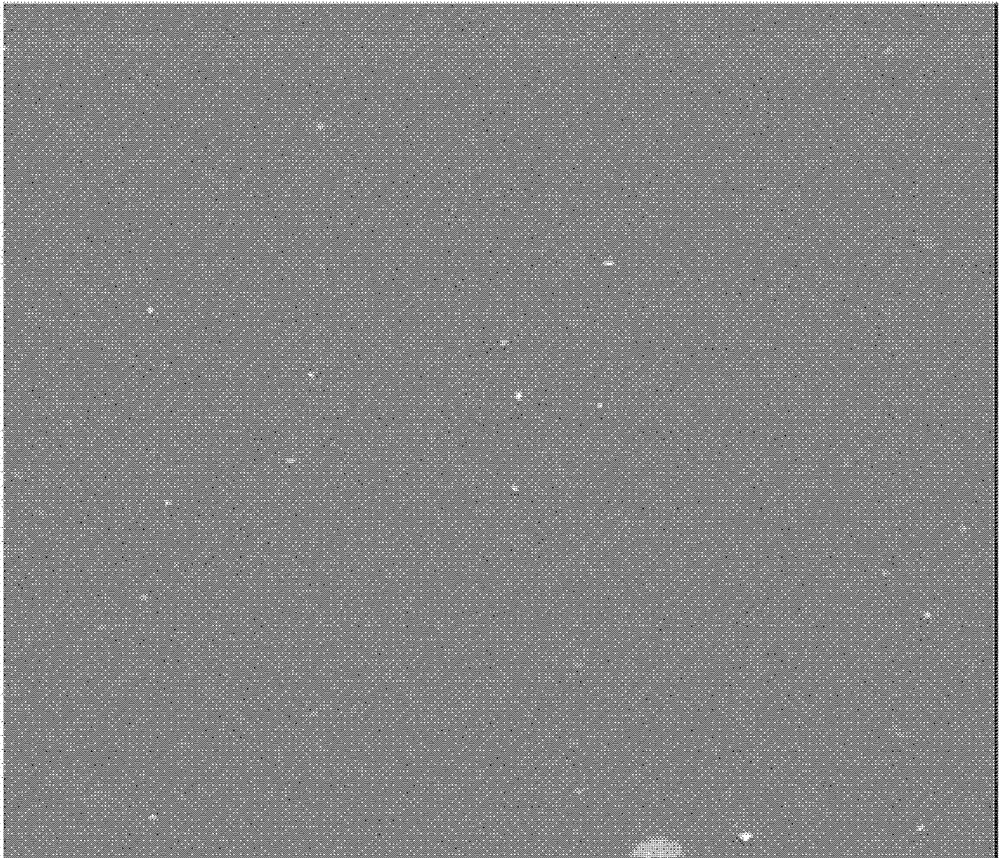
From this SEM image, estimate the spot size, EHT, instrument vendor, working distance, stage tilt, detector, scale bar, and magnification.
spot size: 4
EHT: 5 kV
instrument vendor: FEI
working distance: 11.37 mm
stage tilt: -0.1°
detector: SE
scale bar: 5000 nm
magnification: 10 K X